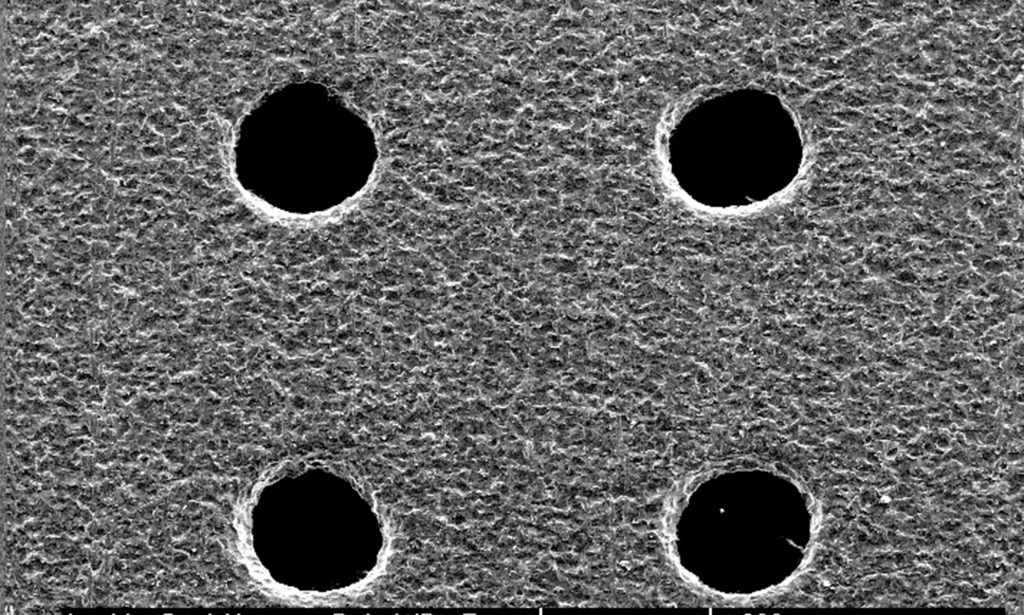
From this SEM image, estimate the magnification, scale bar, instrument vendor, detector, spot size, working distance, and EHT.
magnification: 0.1 K X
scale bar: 200000 nm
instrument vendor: FEI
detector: SE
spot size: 3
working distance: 10.1 mm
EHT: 15 kV